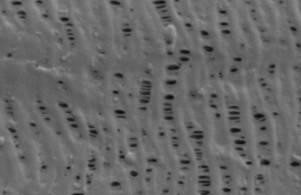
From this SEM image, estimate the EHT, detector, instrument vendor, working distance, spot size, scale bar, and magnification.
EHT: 1 kV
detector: SE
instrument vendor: FEI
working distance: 5.7 mm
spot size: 3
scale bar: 2000 nm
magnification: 16 K X